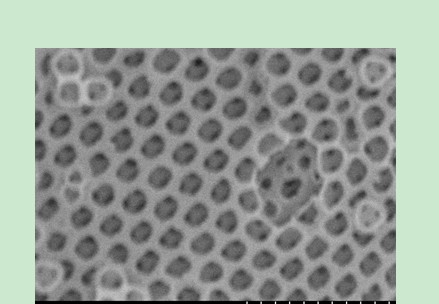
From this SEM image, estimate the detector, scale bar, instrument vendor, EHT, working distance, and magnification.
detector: SE(U)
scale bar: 1000 nm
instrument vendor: Hitachi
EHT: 15 kV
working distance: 6.6 mm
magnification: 50 K X